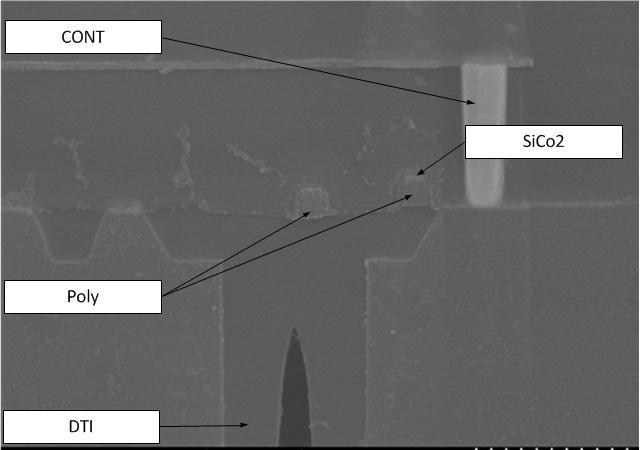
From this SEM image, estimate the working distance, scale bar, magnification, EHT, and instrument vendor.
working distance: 8.1 mm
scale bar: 1000 nm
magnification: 30 K X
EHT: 10 kV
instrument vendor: Hitachi